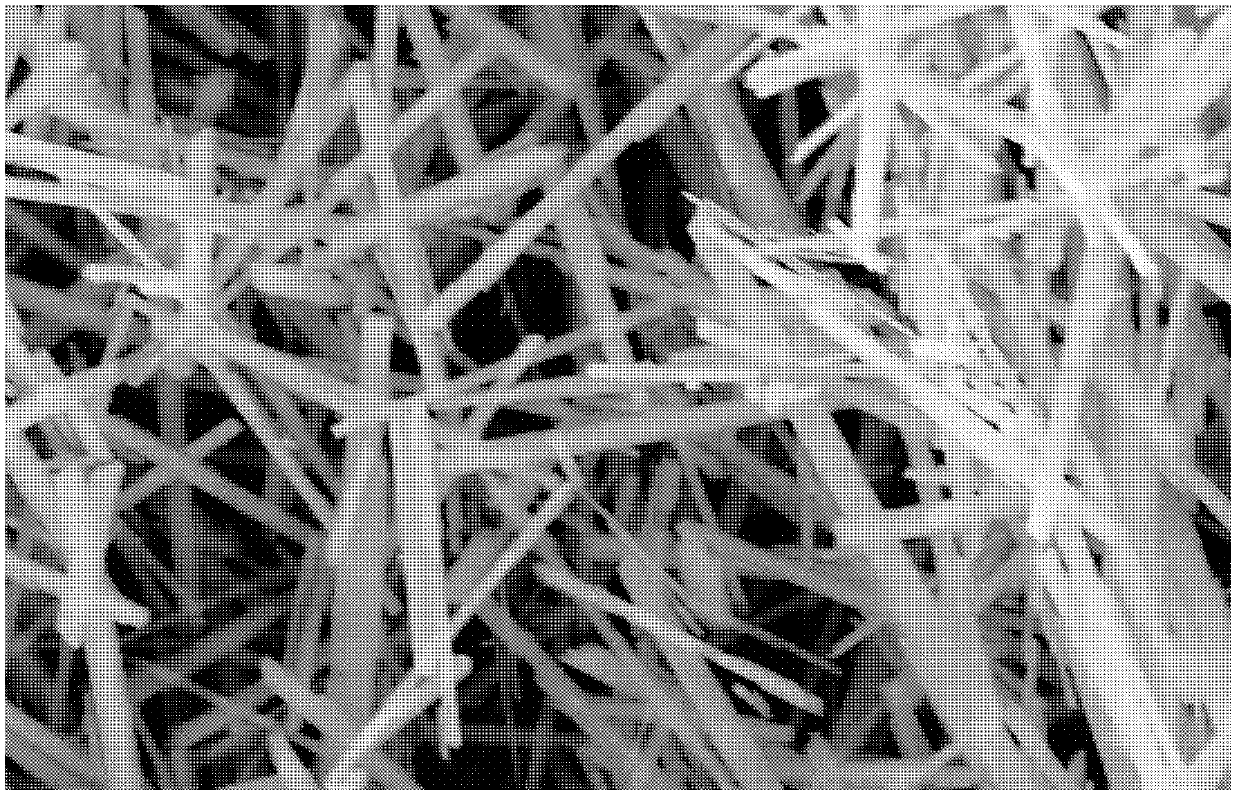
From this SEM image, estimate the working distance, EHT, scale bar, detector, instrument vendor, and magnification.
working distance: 13.6 mm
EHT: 20 kV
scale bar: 20000 nm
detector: SE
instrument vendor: FEI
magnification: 5 K X